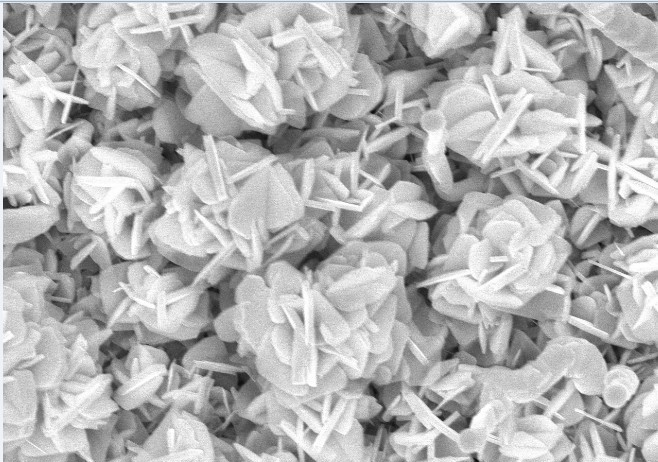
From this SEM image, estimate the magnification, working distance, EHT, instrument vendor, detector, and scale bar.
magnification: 10 K X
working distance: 12 mm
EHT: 15 kV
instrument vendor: Hitachi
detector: SE(U,LA0)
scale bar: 5000 nm